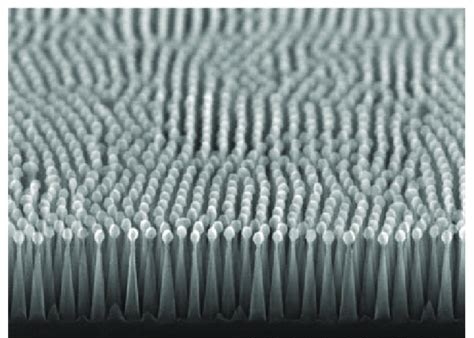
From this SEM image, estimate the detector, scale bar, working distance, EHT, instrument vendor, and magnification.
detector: SE(U,LA0)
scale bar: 500 nm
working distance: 5.7 mm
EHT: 20 kV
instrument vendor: Hitachi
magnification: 100 K X